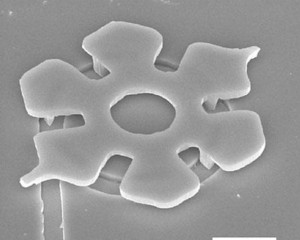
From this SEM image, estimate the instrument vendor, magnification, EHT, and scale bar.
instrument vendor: JEOL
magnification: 4 K X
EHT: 10 kV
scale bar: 7500 nm